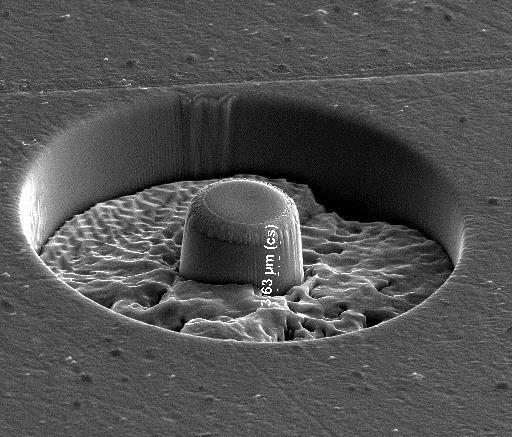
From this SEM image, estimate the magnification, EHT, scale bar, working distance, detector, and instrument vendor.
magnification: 5 K X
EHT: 20 kV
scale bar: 10000 nm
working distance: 10 mm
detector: ETD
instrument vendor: FEI